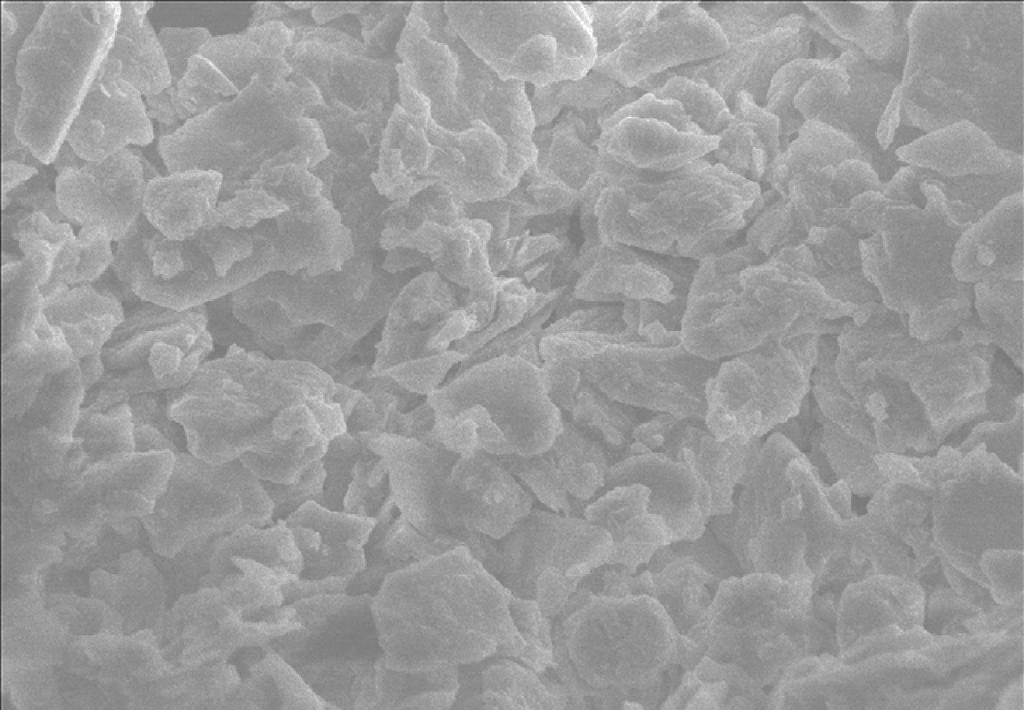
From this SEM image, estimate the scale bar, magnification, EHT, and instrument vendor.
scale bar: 50000 nm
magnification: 1 K X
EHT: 20 kV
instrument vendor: other